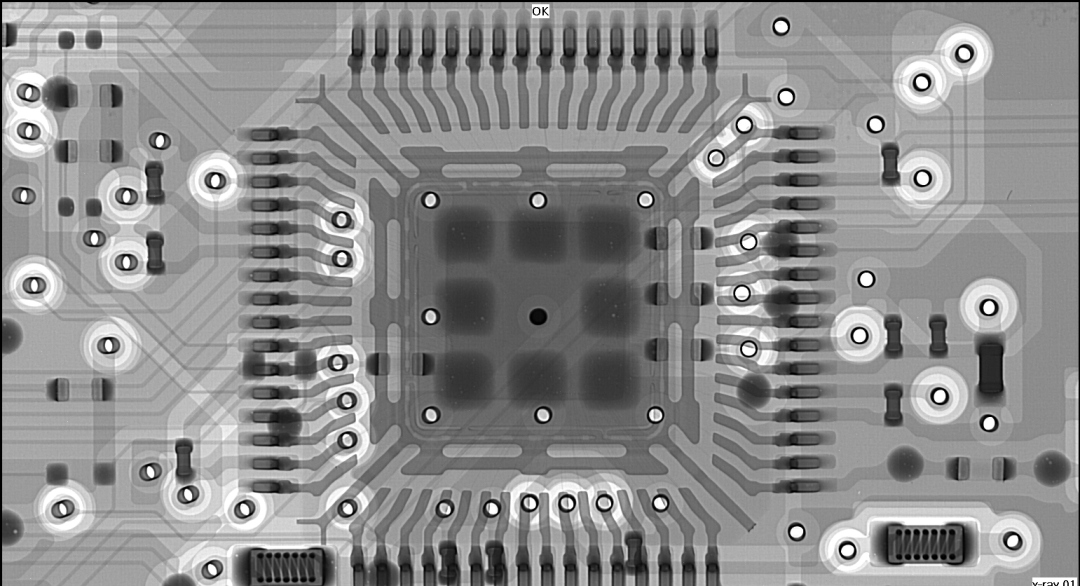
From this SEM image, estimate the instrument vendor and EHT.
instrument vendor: other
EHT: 90 kV